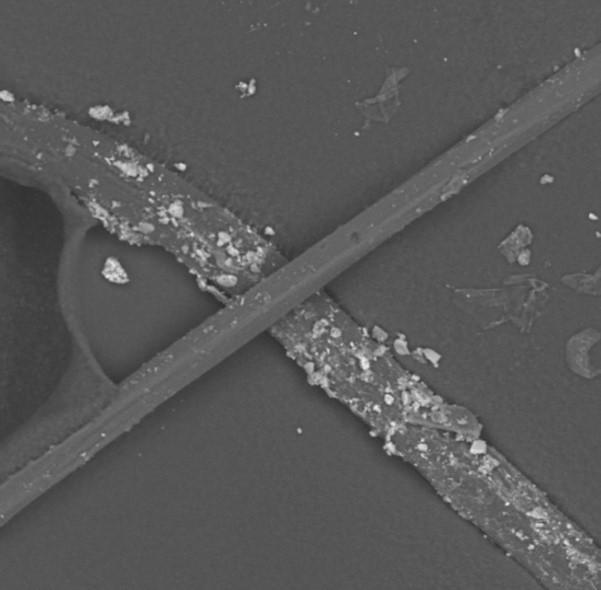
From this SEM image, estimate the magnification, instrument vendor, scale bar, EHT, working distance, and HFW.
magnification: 0.91 K X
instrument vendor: Thermo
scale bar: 80000 nm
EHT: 15 kV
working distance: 11.521 mm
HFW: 295 µm